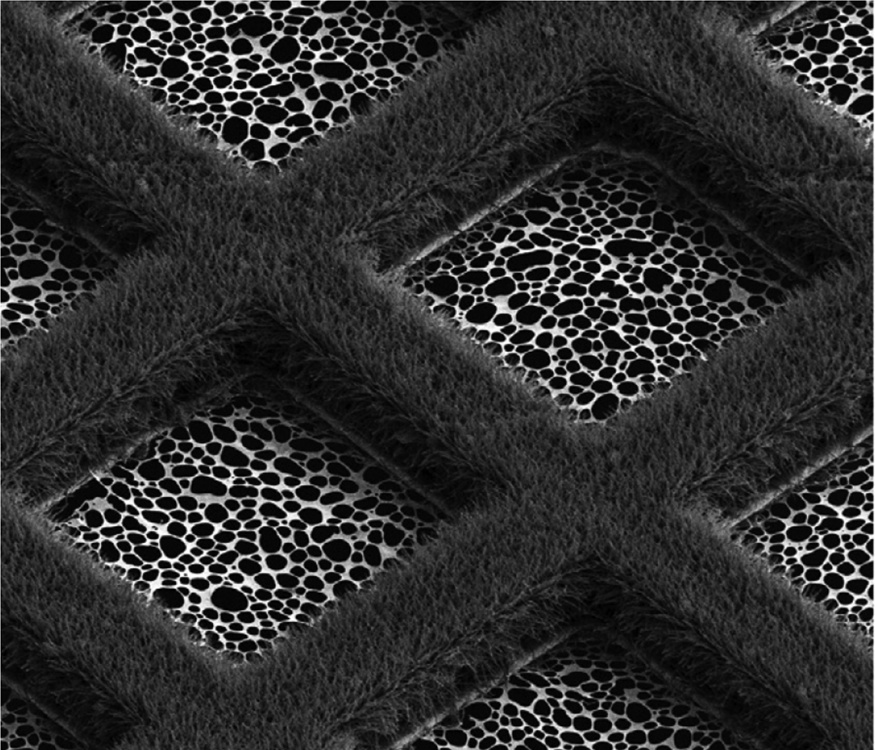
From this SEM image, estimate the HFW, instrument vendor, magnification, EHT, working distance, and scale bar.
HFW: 149 µm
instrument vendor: FEI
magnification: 0.998 K X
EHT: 2 kV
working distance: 7.1 mm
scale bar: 40000 nm